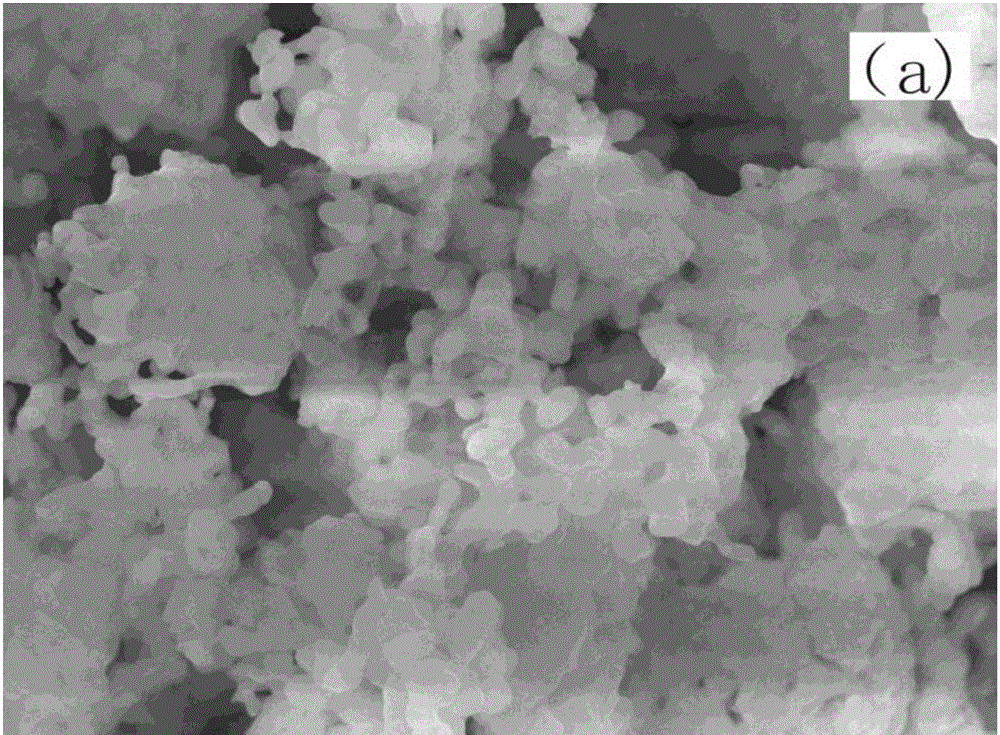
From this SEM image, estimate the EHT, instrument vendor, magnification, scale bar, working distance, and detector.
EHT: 20 kV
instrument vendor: JEOL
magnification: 10 K X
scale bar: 1000 nm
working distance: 10.3 mm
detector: SEI